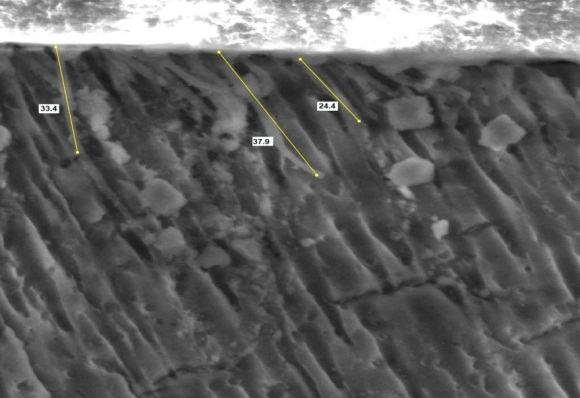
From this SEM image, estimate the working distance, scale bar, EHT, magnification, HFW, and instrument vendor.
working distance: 13.2 mm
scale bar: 50000 nm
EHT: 25 kV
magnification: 2 K X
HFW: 149 µm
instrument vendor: FEI